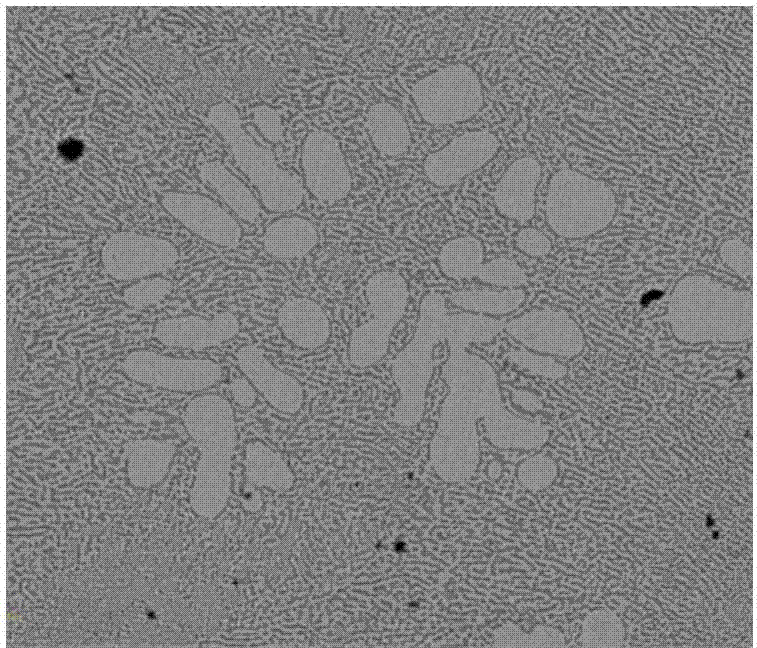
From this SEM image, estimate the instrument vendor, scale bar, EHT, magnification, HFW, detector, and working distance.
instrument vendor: FEI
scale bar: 50000 nm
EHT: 20 kV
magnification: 2.5 K X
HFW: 119 µm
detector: BSED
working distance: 9.1 mm